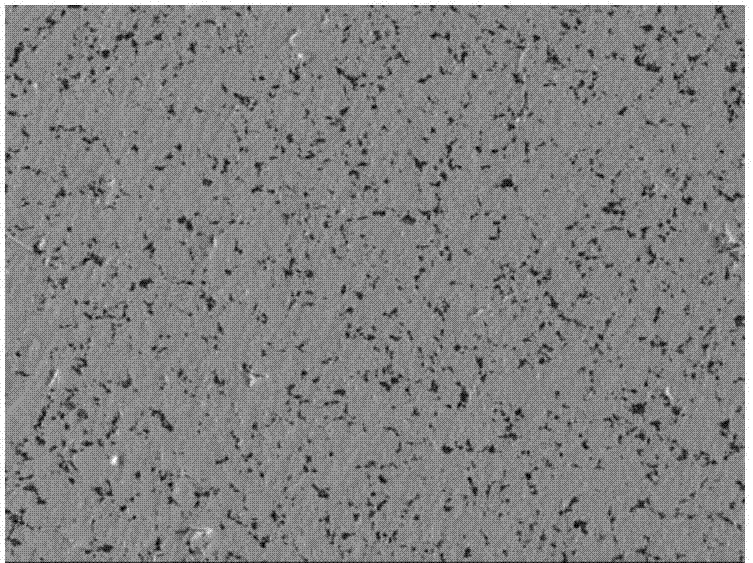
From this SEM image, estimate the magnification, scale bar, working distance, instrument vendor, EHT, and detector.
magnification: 0.5 K X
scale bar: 10000 nm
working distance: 14.7 mm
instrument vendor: JEOL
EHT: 20 kV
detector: LEI